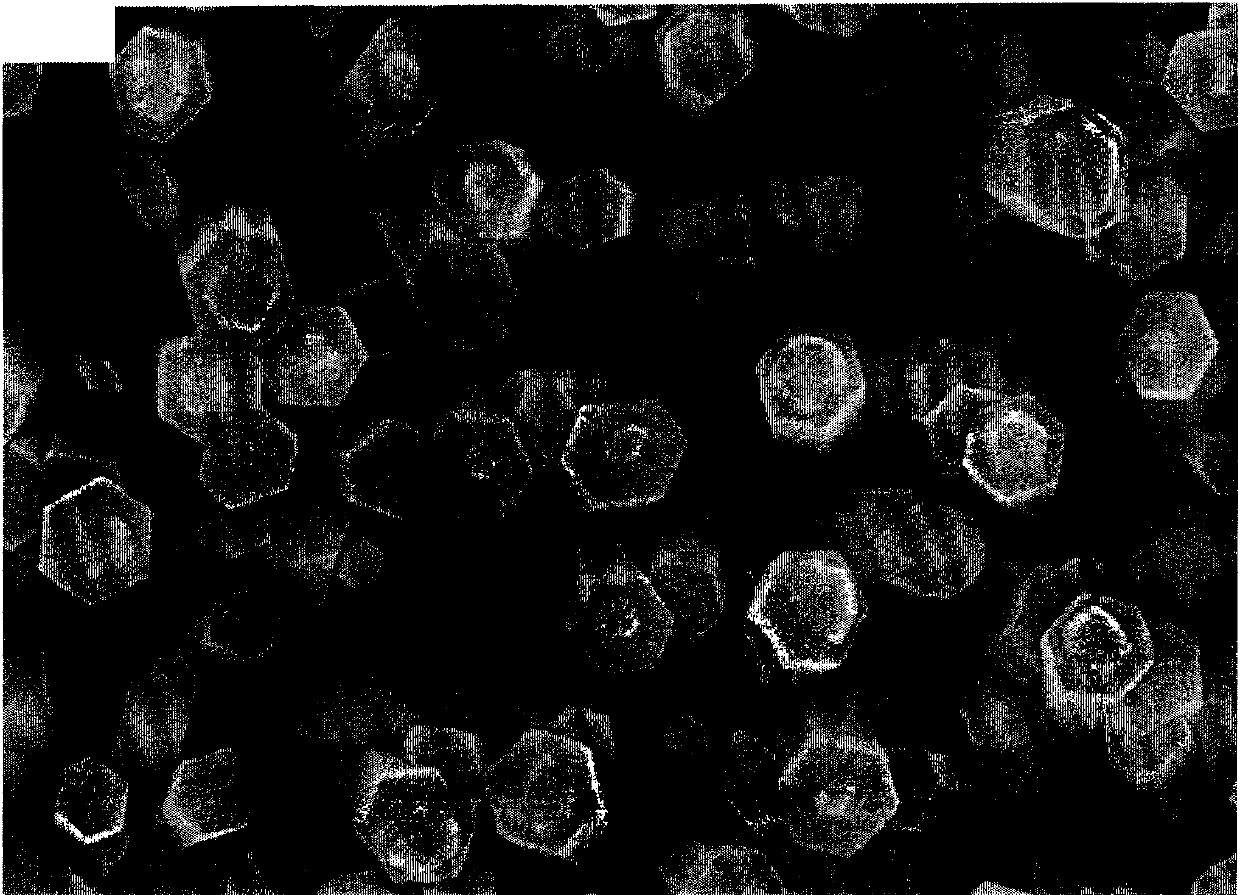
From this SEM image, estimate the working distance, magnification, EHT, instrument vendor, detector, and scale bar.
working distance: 13.8 mm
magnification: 50 K X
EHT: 15 kV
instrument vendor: Hitachi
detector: SE(M)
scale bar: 1000 nm